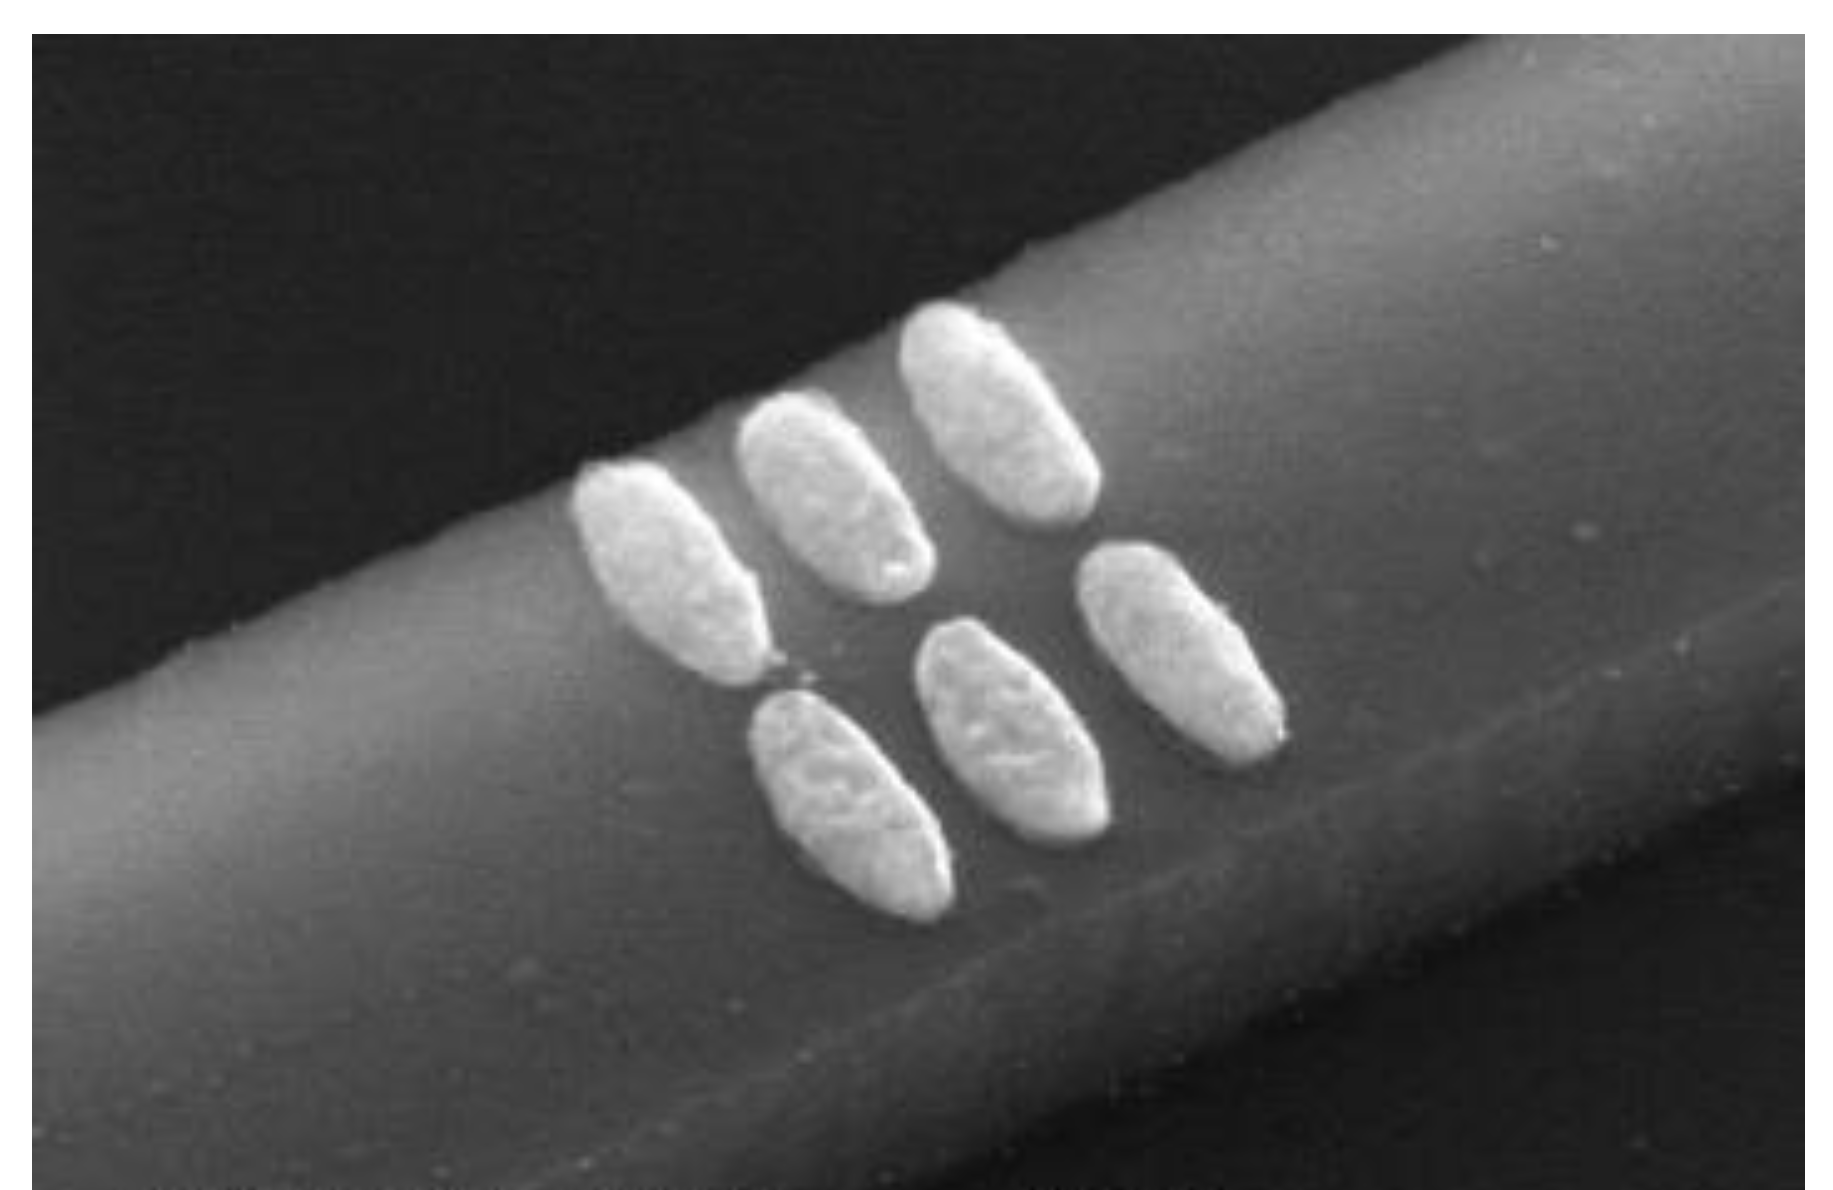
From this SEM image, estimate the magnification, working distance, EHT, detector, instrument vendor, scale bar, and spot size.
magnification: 100 K X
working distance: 5.4 mm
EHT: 15 kV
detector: TLD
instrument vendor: FEI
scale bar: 200 nm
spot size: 2